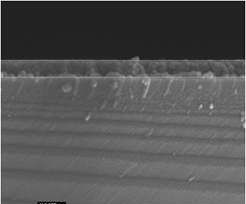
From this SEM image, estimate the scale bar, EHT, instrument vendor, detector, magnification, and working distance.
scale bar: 100 nm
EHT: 10 kV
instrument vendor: JEOL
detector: SEI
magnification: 100 K X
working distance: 6 mm